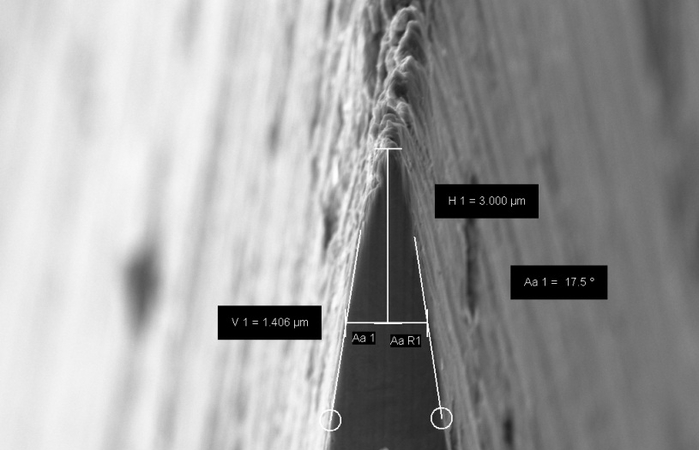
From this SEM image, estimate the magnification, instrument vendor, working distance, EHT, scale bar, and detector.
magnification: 10 K X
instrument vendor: Zeiss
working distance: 6.5 mm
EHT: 5 kV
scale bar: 1000 nm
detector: SE2